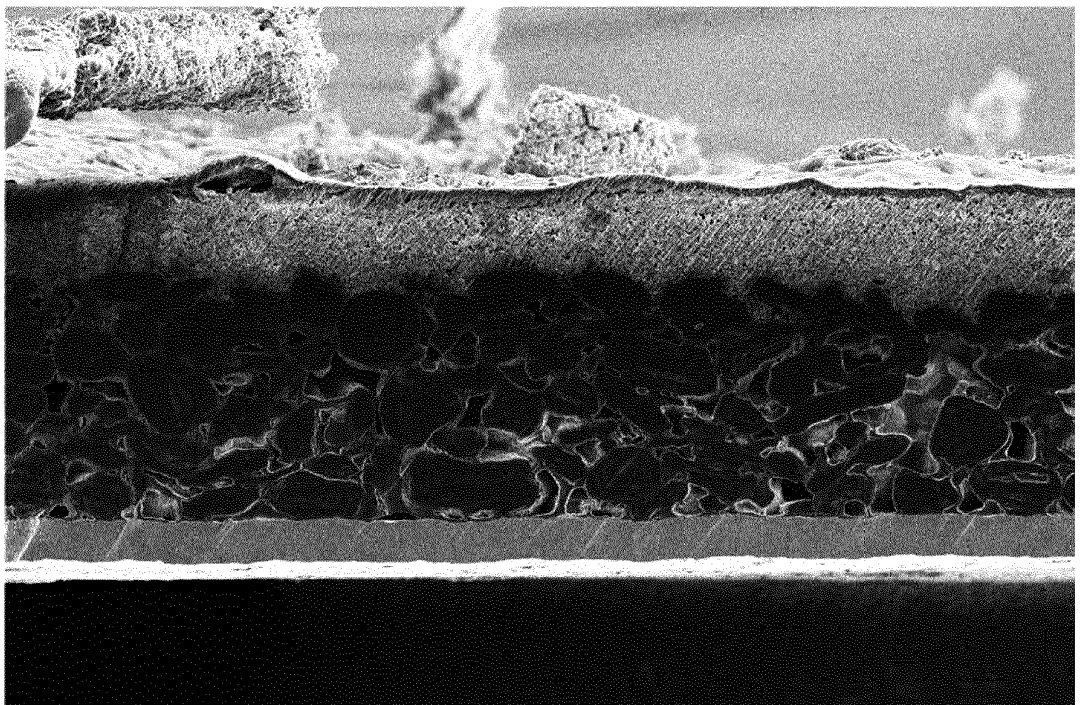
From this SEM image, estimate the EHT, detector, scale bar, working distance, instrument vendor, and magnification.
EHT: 5 kV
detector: ETD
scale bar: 50000 nm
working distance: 11.4 mm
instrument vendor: Thermo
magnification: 0.5 K X